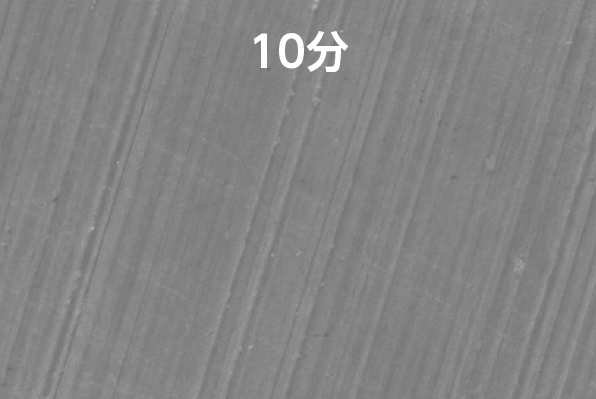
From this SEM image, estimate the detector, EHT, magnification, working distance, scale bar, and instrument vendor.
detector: SED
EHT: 20 kV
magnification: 1 K X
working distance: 10 mm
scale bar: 10000 nm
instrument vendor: JEOL